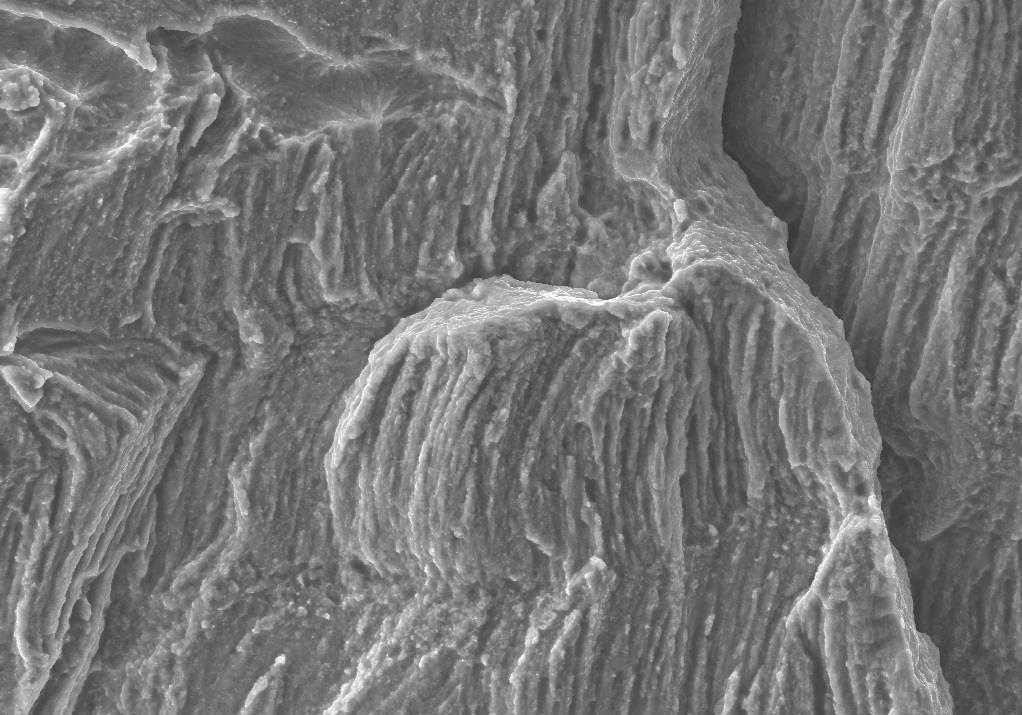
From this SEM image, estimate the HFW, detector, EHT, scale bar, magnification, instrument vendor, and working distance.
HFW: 228.7 µm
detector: SE2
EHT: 20 kV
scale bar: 20000 nm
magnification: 0.5 K X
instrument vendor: Zeiss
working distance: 18 mm